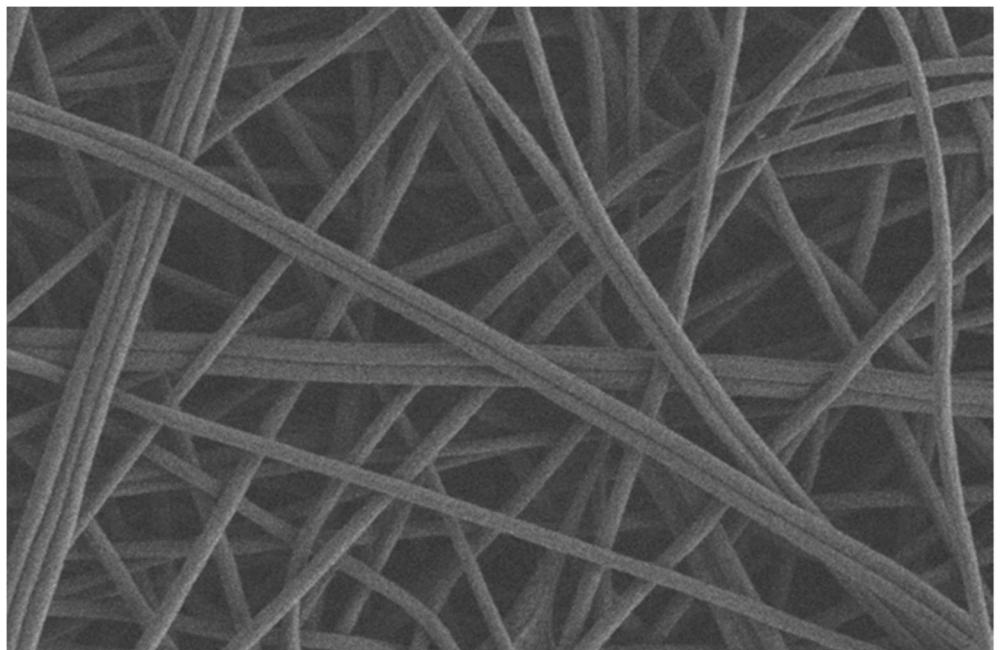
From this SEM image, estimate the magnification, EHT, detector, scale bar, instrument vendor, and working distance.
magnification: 5 K X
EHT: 2 kV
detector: SE2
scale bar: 2000 nm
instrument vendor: Zeiss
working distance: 7.6 mm